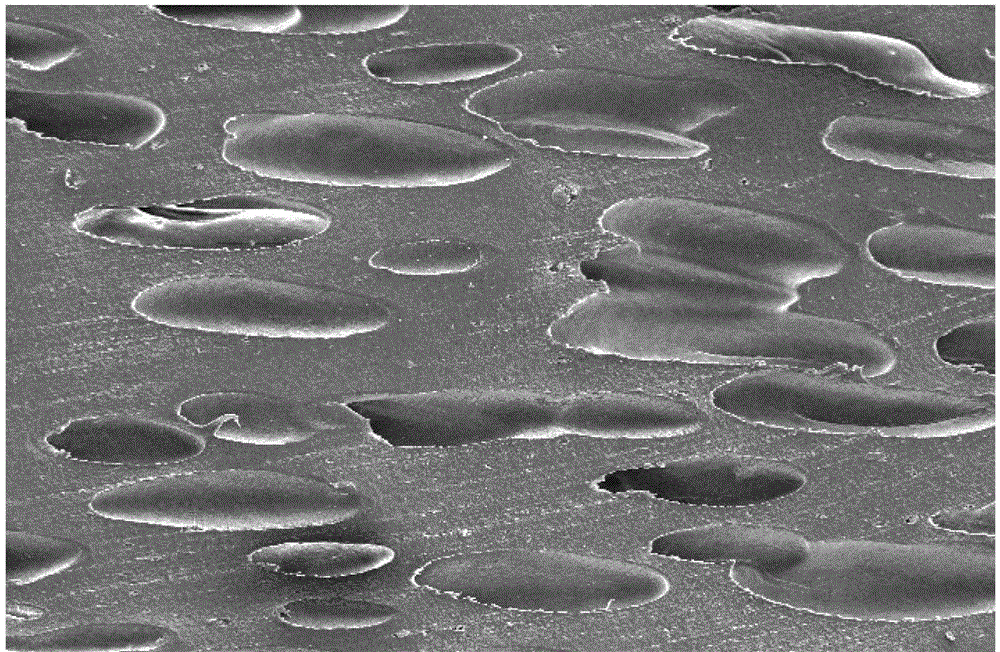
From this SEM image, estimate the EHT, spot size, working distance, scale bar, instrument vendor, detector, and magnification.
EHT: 5 kV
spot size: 3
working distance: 8 mm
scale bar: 200000 nm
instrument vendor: FEI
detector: SE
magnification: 0.1 K X